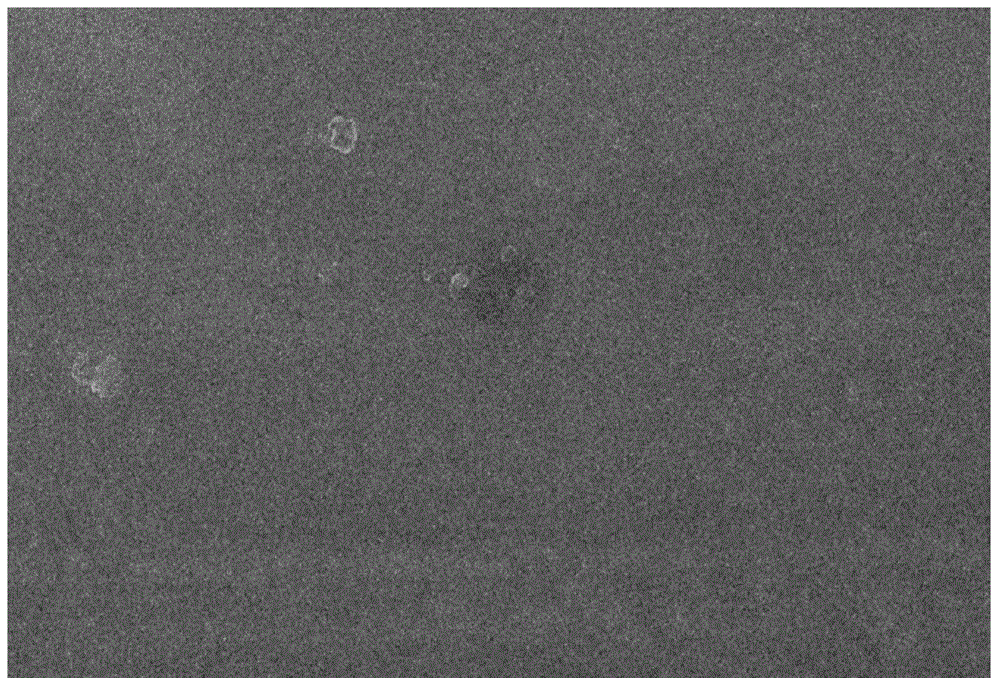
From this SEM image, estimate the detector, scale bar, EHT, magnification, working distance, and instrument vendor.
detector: SEM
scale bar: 1000 nm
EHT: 15 kV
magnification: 5 K X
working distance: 7.2 mm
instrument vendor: JEOL